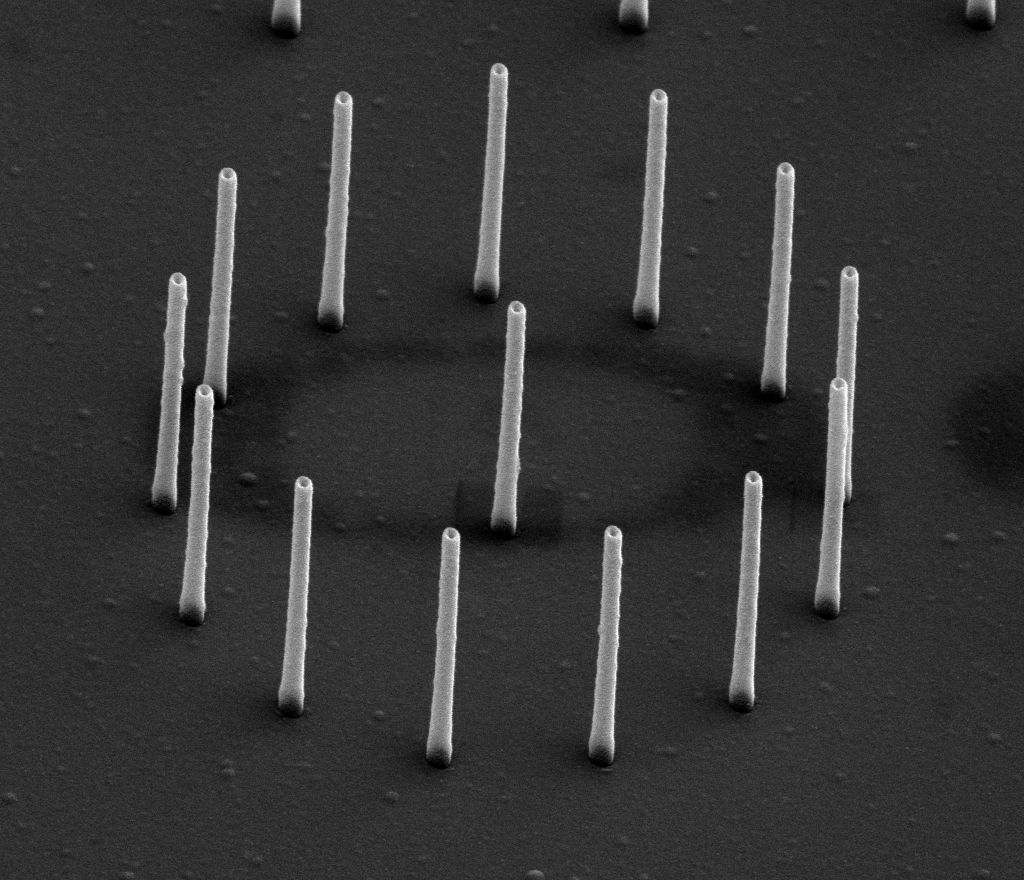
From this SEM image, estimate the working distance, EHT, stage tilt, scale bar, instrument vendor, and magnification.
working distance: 4 mm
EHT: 20 kV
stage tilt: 45°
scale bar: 2000 nm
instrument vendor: FEI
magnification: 25 K X